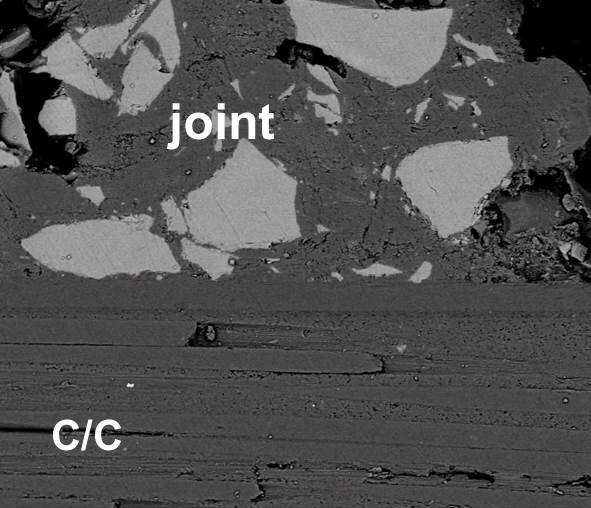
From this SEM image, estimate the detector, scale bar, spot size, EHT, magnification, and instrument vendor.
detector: BSED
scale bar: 50000 nm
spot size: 3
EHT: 10 kV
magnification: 1.6 K X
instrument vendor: FEI